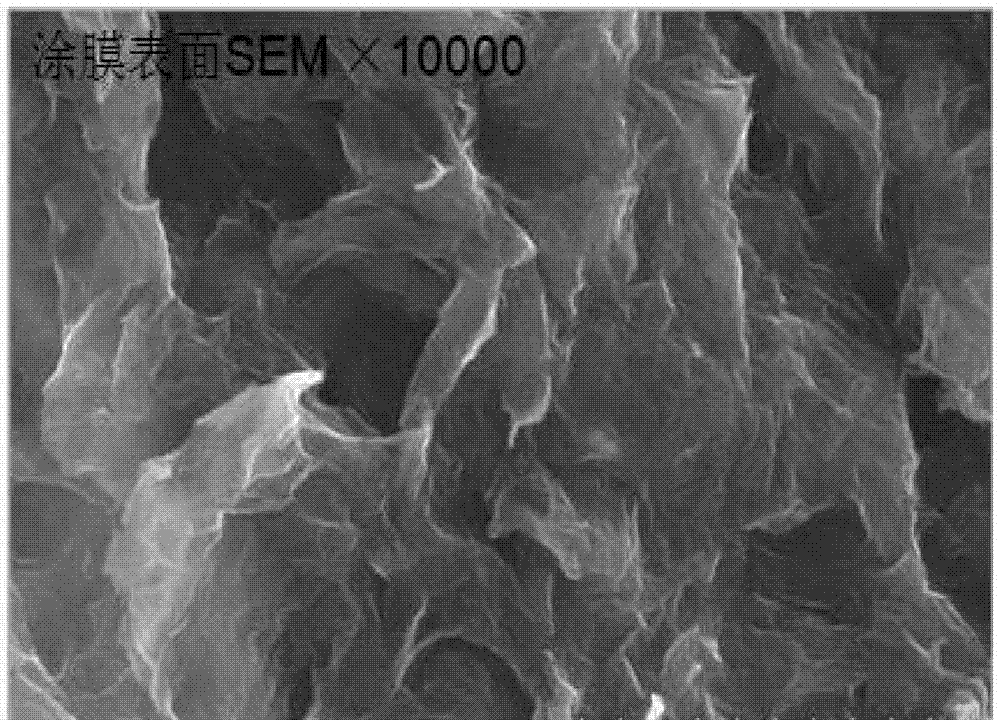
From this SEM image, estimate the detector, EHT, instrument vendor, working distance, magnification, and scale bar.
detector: SE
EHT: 10 kV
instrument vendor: Hitachi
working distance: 7.8 mm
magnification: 10 K X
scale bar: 5000 nm